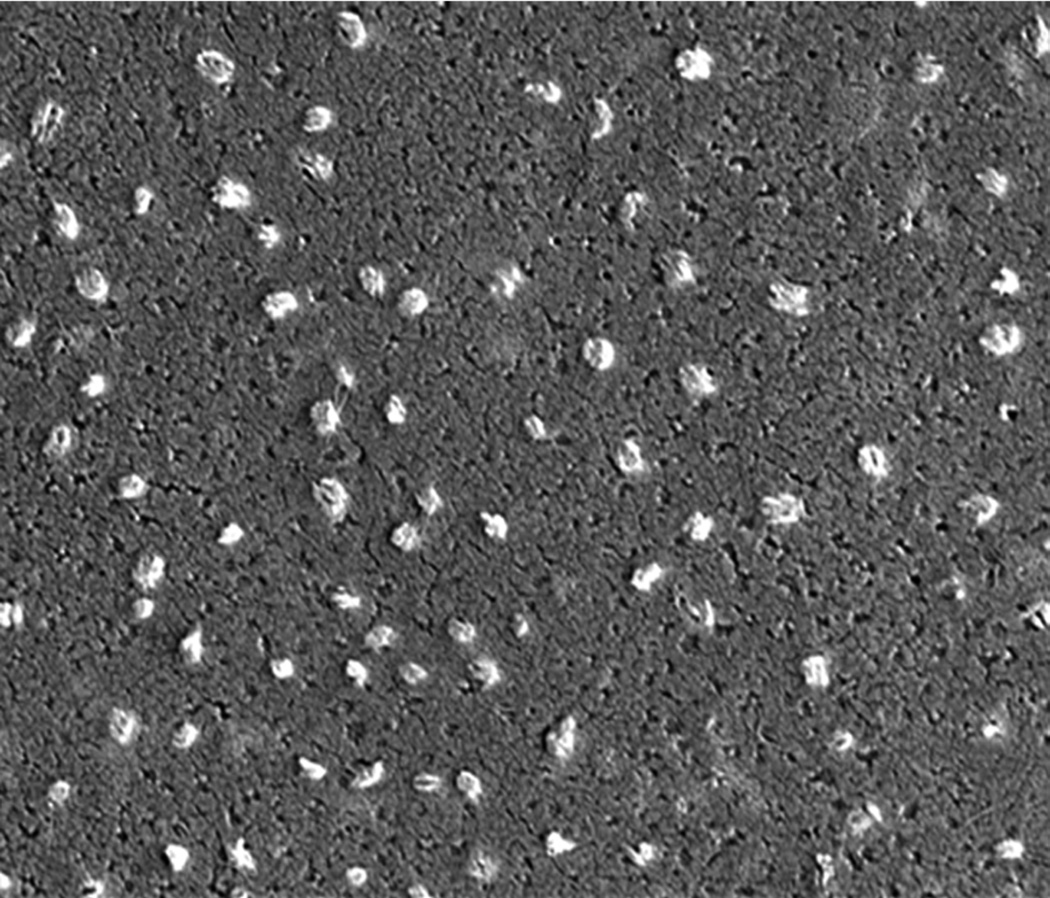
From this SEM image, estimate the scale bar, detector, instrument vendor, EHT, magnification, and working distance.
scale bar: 3000 nm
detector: ETD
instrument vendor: FEI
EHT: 15 kV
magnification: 15 K X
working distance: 5 mm